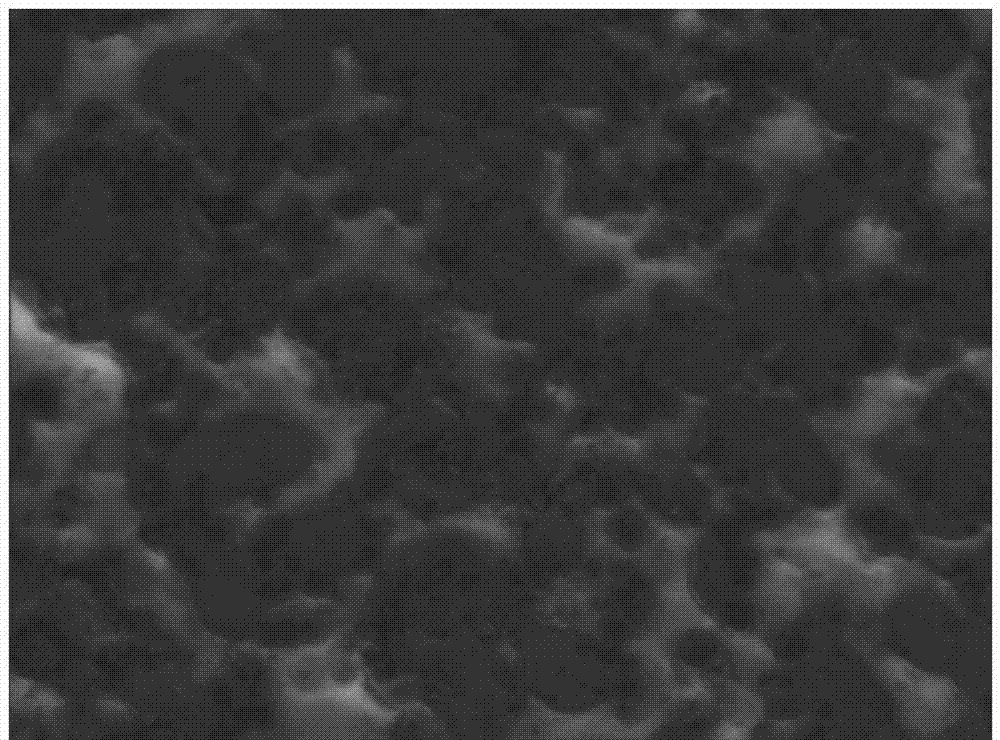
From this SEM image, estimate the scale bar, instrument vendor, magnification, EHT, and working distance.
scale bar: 2000 nm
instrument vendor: Zeiss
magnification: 5 K X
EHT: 5 kV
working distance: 16.26 mm